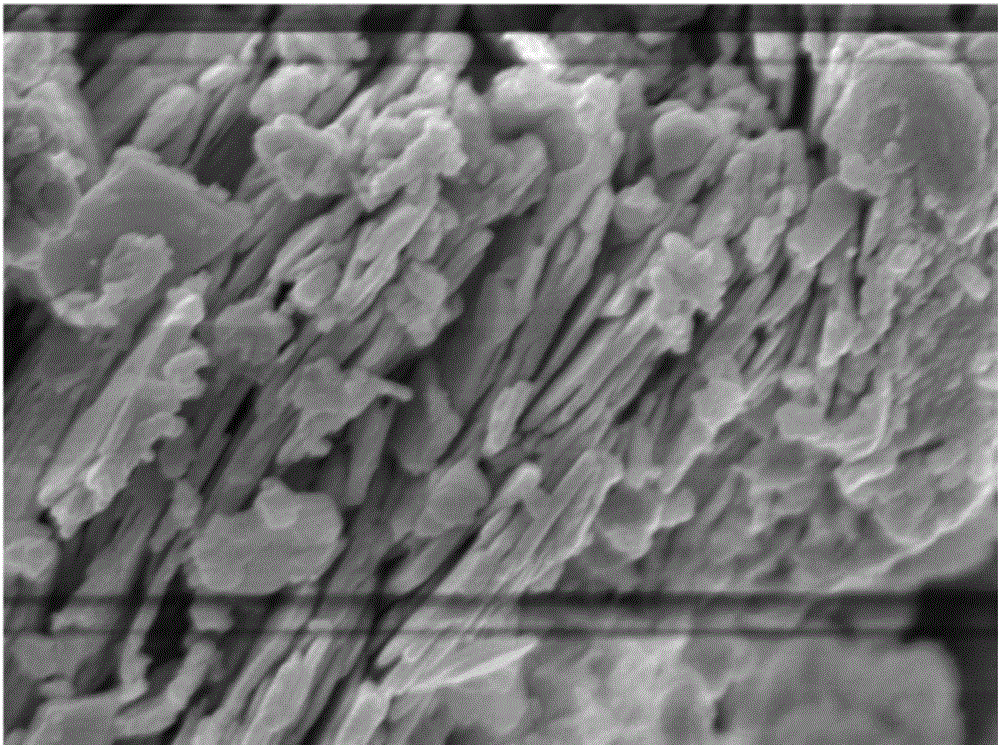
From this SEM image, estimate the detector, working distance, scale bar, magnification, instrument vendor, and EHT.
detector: SEM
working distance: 8.3 mm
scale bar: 100 nm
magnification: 30 K X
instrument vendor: JEOL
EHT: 5 kV